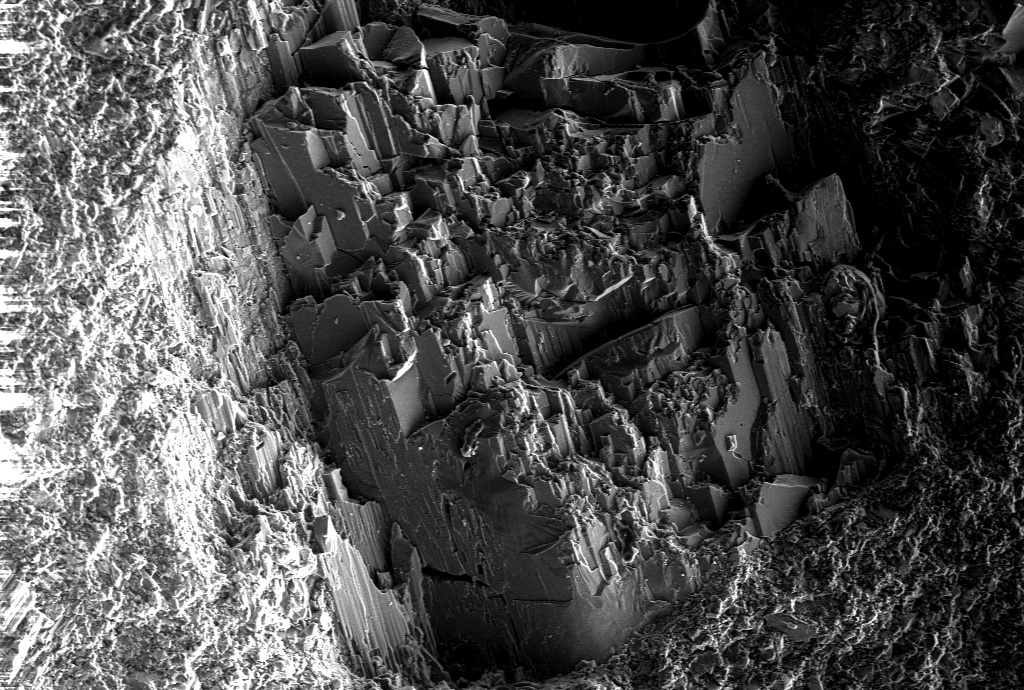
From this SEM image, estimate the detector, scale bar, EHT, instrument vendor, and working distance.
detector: C2D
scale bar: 40000 nm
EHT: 10 kV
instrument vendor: Zeiss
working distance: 9.07 mm